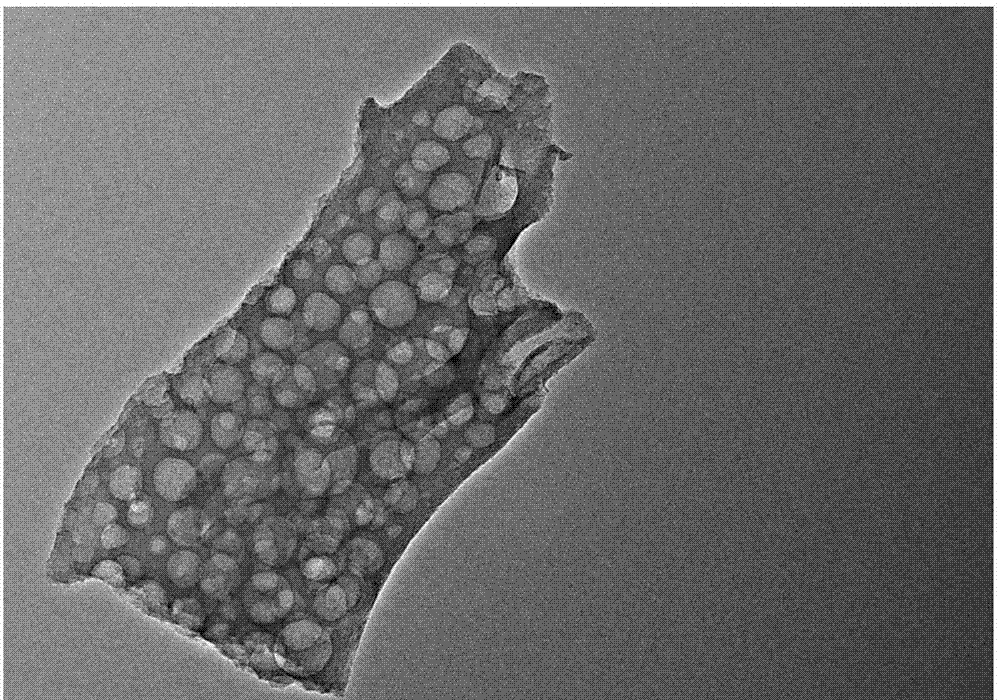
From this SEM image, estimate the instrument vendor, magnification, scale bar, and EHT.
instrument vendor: FEI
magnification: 29 K X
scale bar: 200 nm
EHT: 200 kV